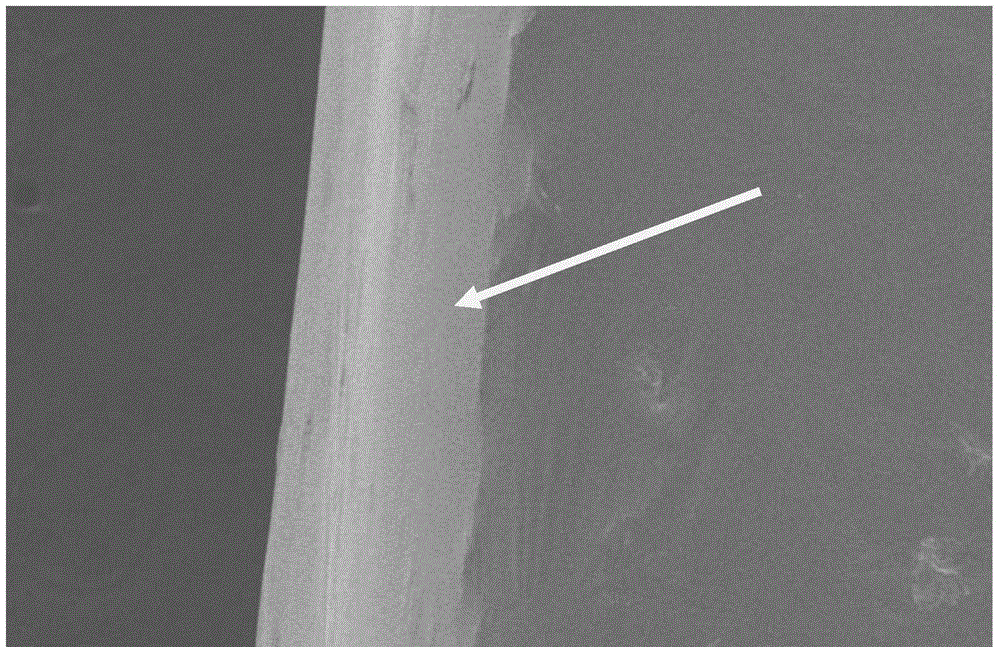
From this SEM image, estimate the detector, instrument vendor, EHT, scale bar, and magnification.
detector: SE(M)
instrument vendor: Hitachi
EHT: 10 kV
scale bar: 200000 nm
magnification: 0.25 K X